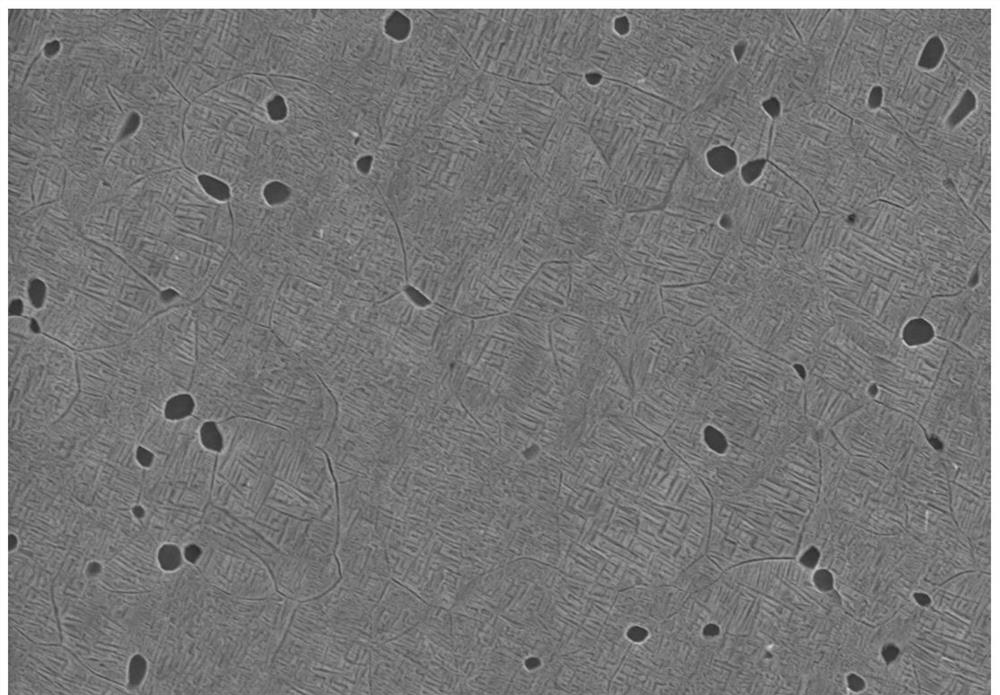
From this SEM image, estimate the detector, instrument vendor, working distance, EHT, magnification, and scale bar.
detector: SE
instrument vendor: Hitachi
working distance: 10.5 mm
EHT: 15 kV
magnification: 5 K X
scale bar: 10000 nm